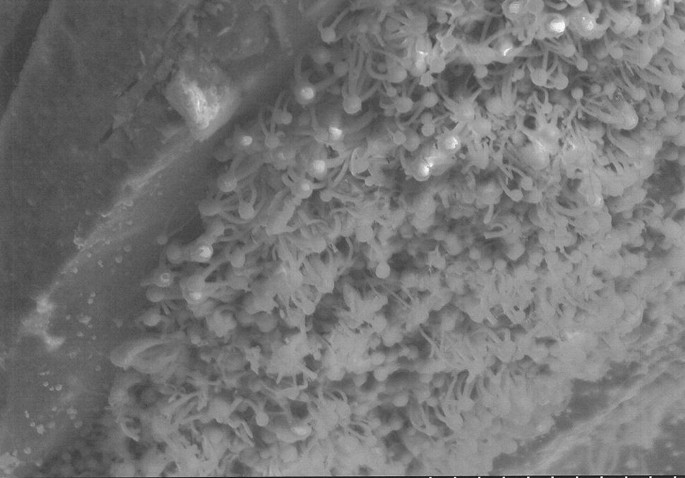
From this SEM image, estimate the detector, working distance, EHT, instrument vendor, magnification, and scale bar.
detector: SE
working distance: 15.1 mm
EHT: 15 kV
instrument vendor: Hitachi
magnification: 1.5 K X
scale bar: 30000 nm